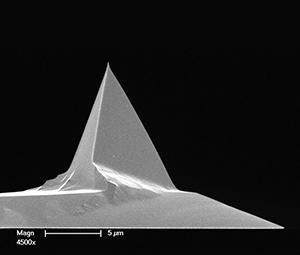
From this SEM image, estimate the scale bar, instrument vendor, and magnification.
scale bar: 5000 nm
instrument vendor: FEI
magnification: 4.5 K X